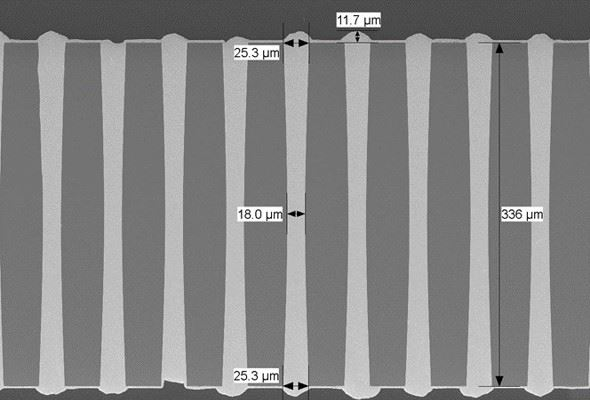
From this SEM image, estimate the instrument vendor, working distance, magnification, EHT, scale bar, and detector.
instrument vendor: Hitachi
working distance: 8.3 mm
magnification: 0.22 K X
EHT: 10 kV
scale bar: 200000 nm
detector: LM(L)